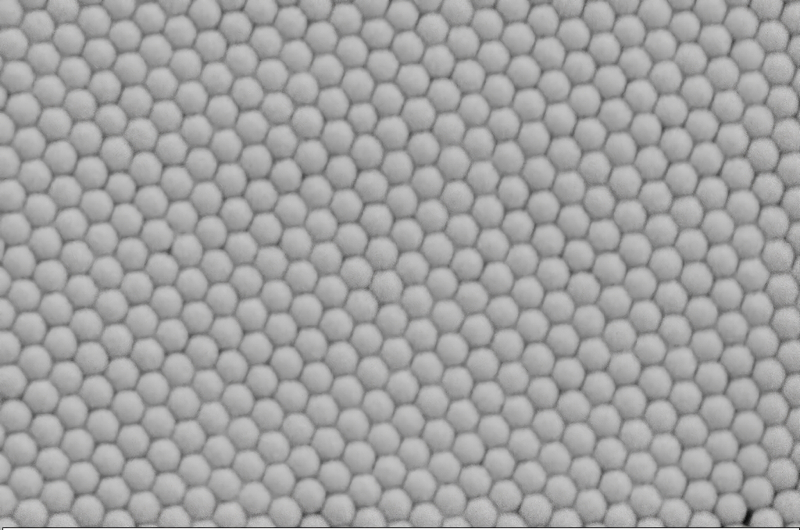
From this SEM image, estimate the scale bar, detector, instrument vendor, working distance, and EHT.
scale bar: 1000 nm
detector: SE2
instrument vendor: Zeiss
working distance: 7.9 mm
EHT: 3 kV